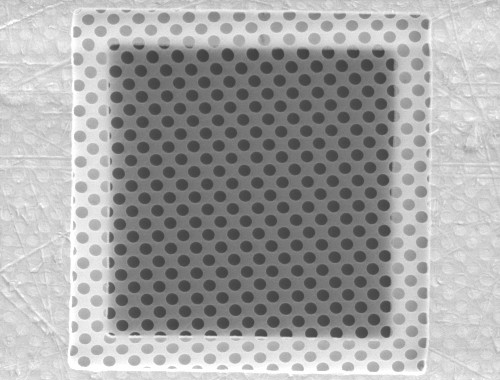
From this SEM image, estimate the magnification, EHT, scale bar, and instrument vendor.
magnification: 1.65 K X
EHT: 5 kV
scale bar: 20000 nm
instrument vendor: other